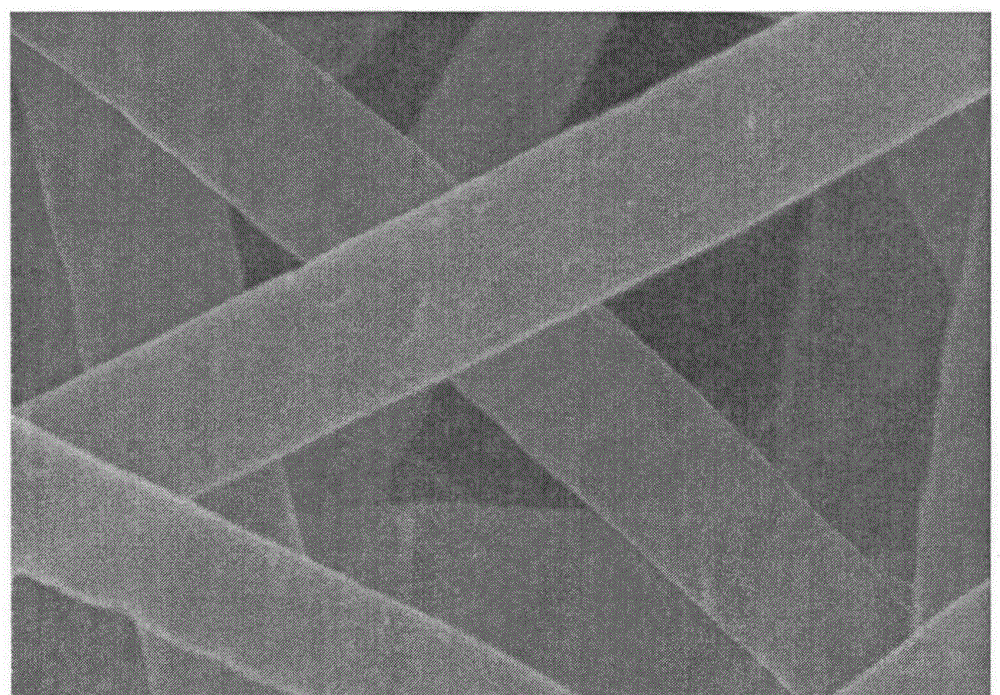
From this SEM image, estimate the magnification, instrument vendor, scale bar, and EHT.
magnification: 100 K X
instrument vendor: Hitachi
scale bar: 500 nm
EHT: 5 kV